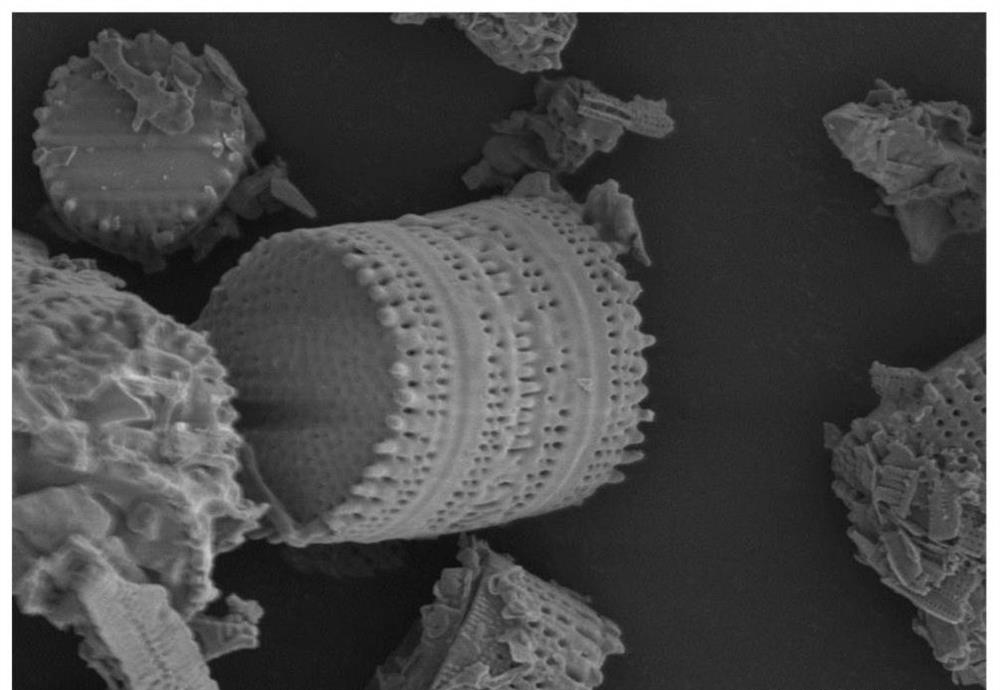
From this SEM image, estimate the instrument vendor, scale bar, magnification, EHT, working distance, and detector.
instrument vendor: Hitachi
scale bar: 10000 nm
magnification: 3 K X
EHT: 3 kV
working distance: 9.1 mm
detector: SE(UL)